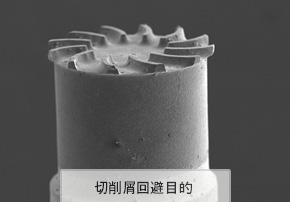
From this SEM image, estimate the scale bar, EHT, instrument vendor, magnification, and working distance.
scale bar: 200000 nm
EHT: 12 kV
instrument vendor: Hitachi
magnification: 0.05 K X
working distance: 8.4 mm